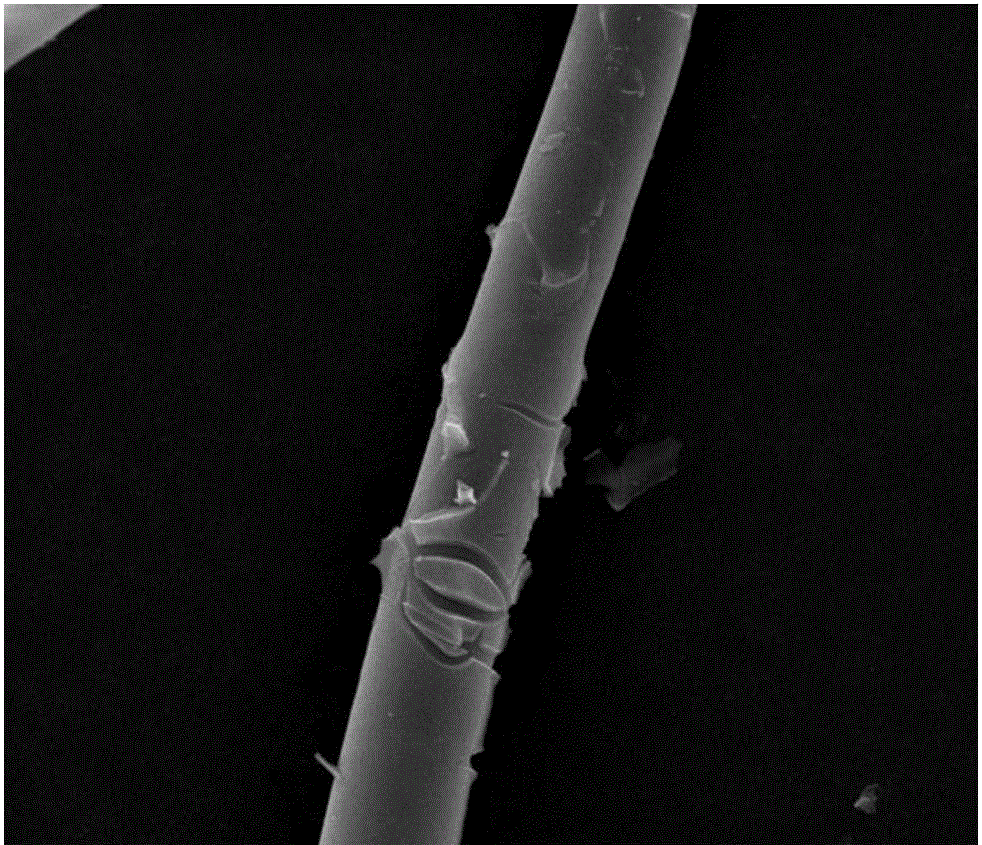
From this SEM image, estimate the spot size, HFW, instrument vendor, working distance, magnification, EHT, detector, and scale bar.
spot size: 3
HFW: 59.7 µm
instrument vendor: FEI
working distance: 12 mm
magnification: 5 K X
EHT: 20 kV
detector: ETD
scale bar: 20000 nm